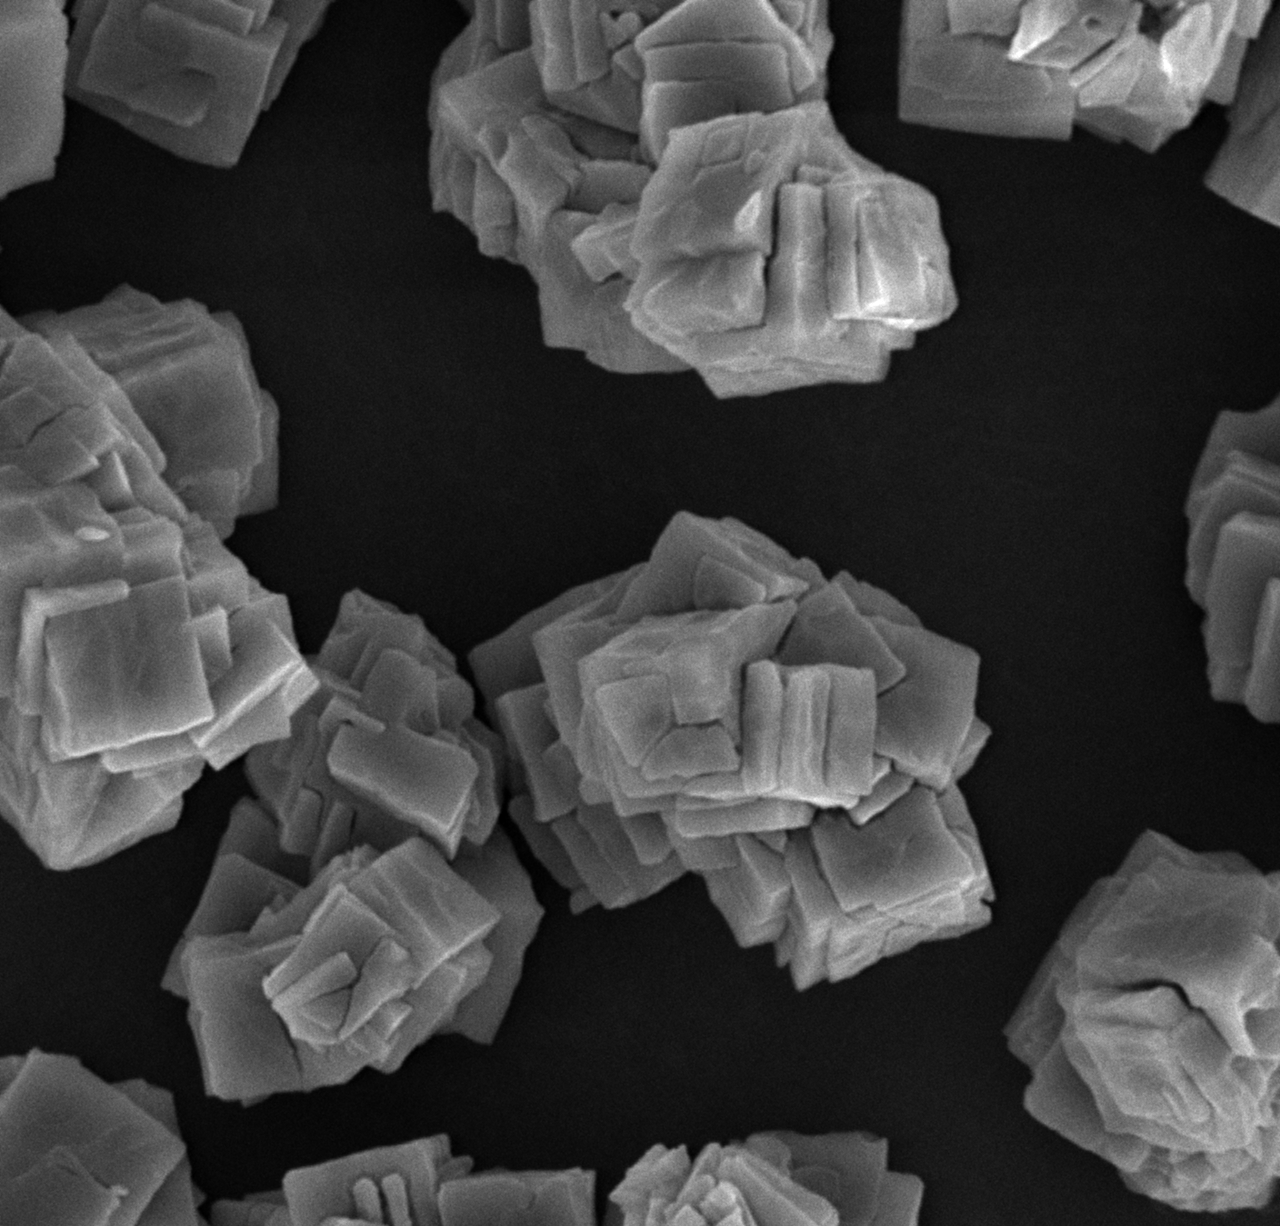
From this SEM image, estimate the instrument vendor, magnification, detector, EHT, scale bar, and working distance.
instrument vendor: other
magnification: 6 K X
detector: SE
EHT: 15 kV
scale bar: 5000 nm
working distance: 10.1 mm